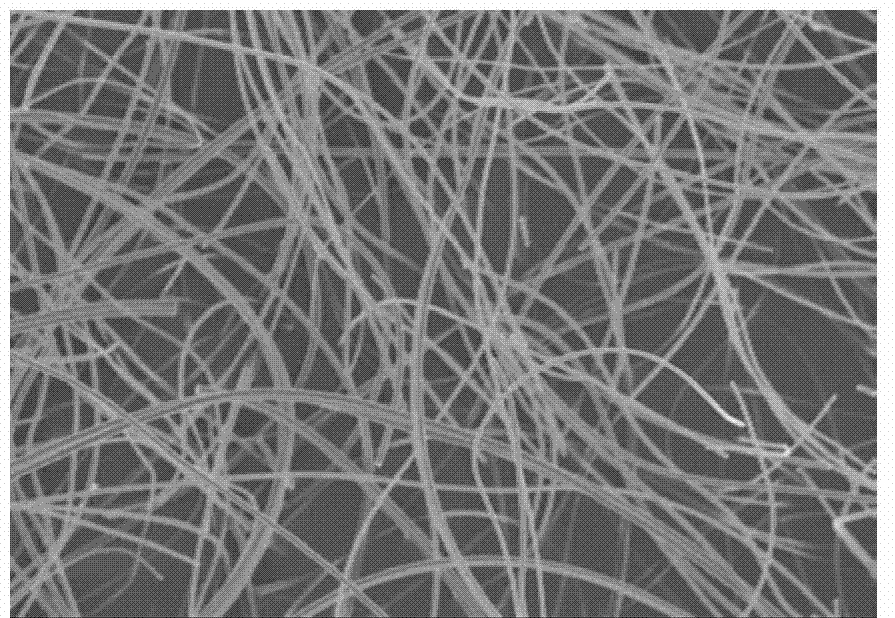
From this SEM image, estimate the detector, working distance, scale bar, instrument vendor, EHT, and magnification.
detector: SE(M)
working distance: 8.3 mm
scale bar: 10000 nm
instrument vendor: Hitachi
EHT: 5 kV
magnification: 3 K X